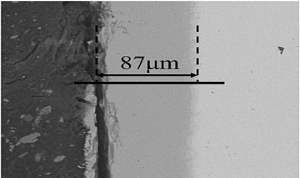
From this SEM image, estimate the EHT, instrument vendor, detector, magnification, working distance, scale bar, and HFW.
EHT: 20 kV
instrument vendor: FEI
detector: vCD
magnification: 0.5 K X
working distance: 10.4 mm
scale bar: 100000 nm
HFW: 254 µm